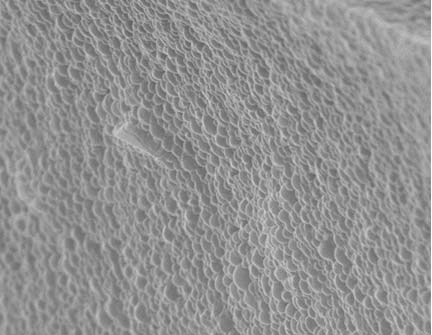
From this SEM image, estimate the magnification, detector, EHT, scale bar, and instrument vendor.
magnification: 30 K X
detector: SE1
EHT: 20 kV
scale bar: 1000 nm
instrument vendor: Zeiss